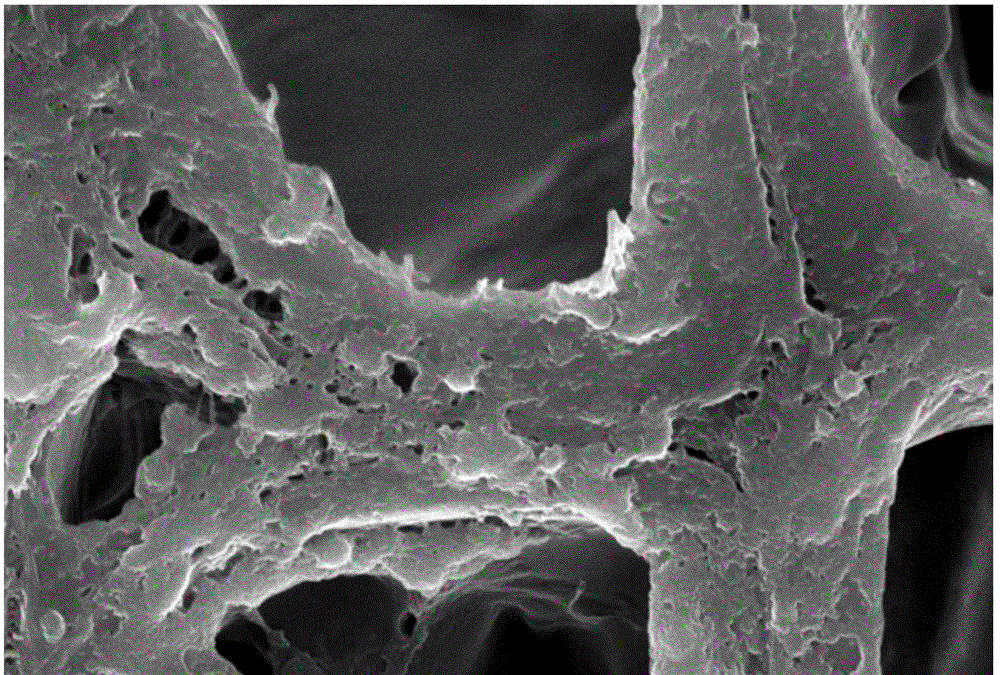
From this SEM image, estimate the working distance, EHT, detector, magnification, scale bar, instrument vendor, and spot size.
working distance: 13 mm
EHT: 20 kV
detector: SEI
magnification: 5 K X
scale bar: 5000 nm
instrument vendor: JEOL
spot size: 40